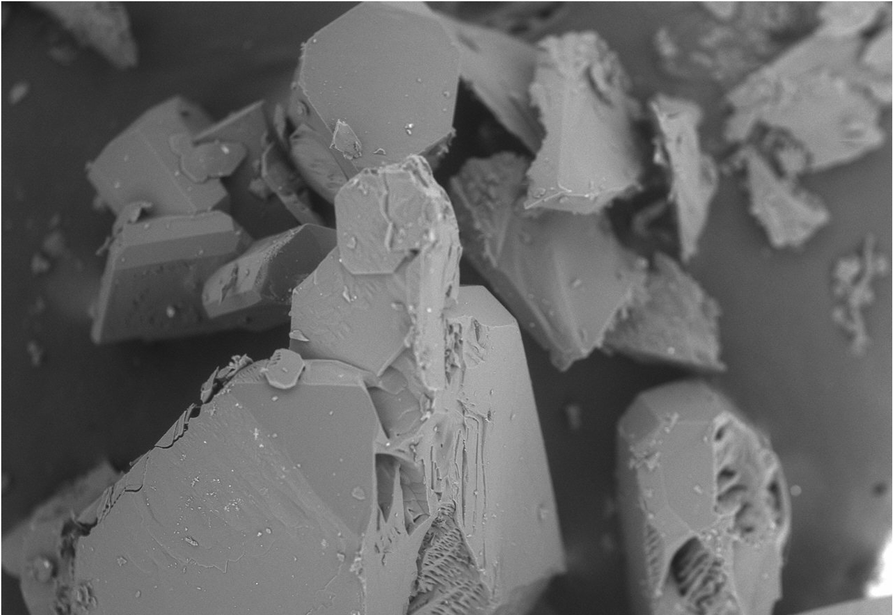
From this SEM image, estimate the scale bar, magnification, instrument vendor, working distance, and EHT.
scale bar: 500000 nm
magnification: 0.085 K X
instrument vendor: Hitachi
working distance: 4.7 mm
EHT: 15 kV